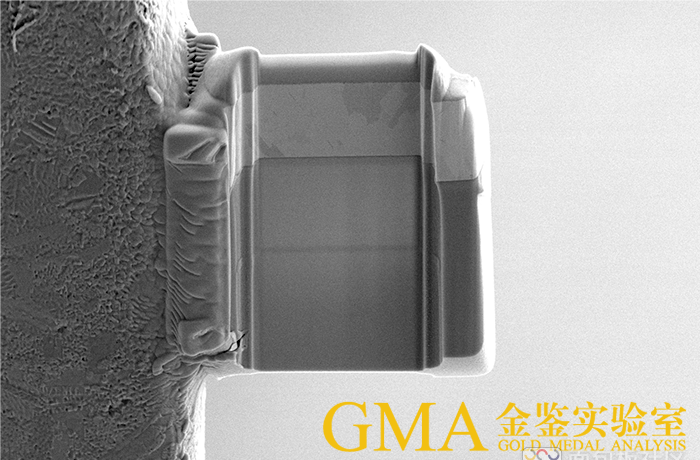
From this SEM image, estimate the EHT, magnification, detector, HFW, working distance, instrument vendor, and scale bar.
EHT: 5 kV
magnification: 5 K X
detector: ETD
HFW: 25.4 µm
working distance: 6.9 mm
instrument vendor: FEI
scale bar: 10000 nm